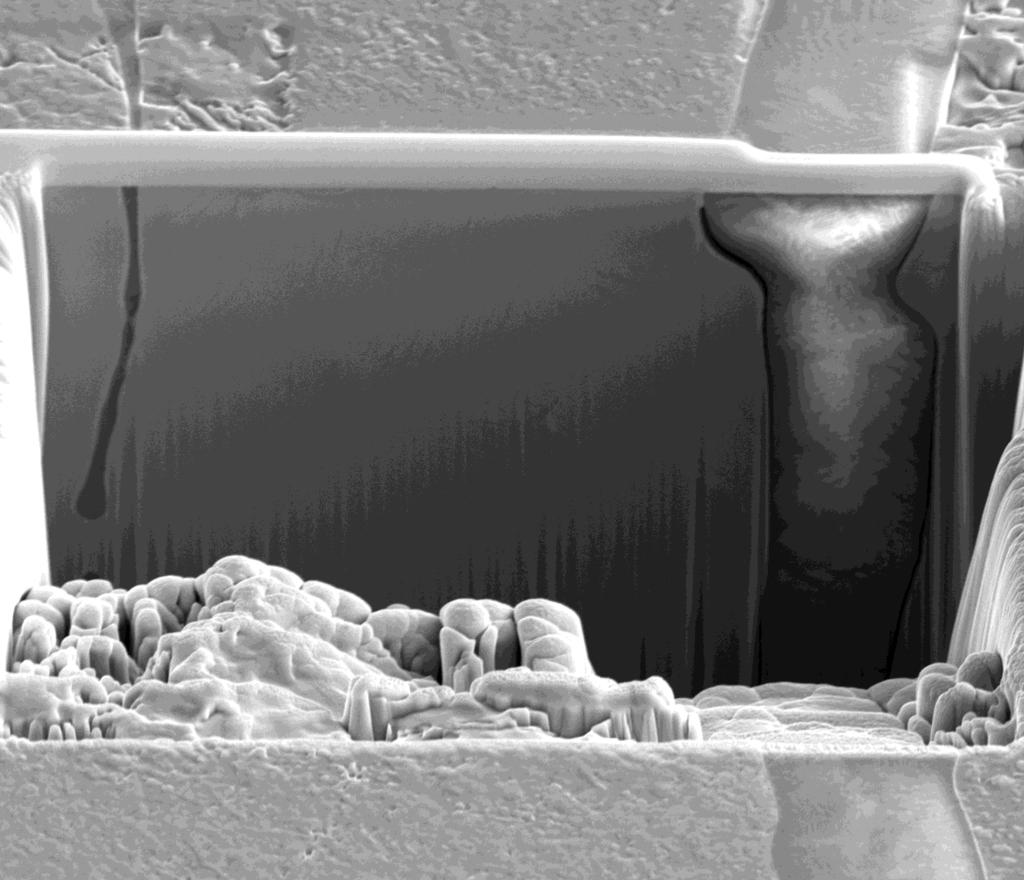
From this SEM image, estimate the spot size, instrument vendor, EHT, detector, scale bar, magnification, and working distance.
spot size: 4.5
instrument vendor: FEI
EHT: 10 kV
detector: ETD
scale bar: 10000 nm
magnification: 5 K X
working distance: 10 mm